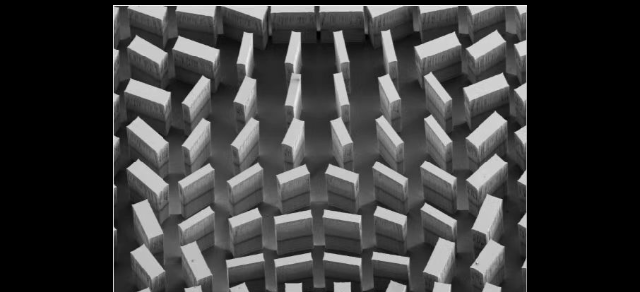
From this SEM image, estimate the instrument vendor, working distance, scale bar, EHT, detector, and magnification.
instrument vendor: Hitachi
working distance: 8 mm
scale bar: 500000 nm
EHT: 3 kV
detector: SE(M)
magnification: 0.1 K X